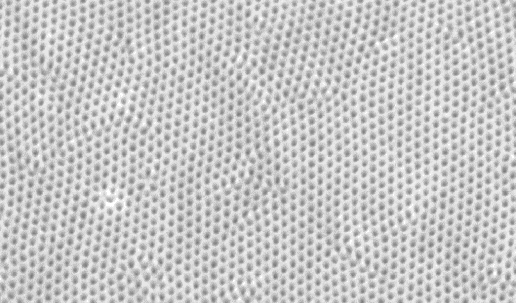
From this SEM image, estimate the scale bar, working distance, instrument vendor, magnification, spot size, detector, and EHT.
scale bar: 2000 nm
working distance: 9.6 mm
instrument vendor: FEI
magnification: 15.561 K X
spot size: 2.9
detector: SE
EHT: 10 kV